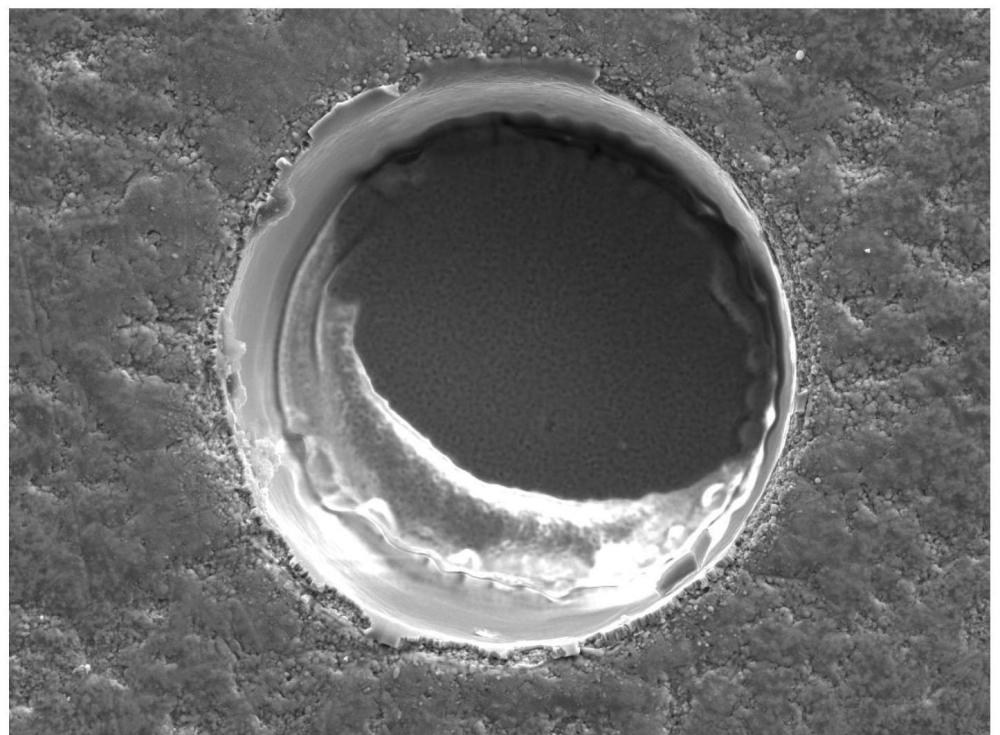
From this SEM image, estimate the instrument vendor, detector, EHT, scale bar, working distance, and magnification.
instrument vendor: JEOL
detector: LED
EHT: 10 kV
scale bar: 10000 nm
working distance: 10 mm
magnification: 1.2 K X